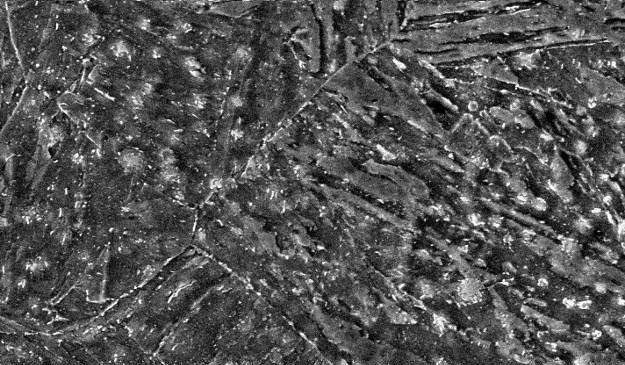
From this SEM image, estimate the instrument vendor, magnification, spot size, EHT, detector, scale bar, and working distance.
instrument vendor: FEI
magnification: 3 K X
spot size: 3.8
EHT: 20 kV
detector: SE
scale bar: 5000 nm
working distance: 10.5 mm